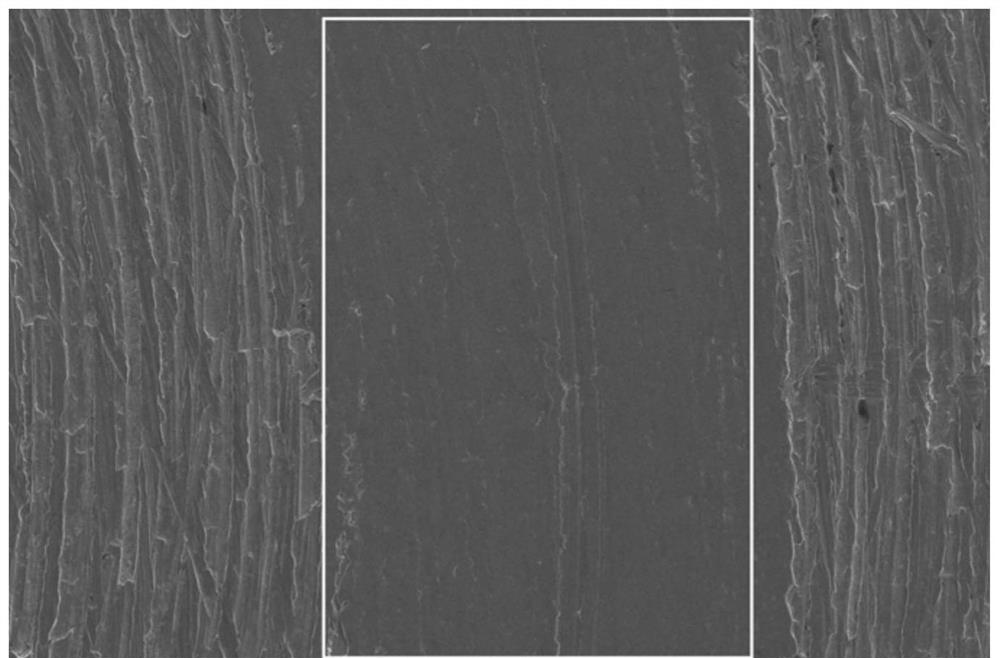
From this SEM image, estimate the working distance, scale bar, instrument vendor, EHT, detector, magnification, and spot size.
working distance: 11 mm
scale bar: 400000 nm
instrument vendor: FEI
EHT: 20 kV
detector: ETD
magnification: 0.4 K X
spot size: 3.5